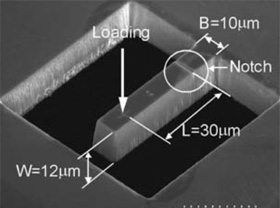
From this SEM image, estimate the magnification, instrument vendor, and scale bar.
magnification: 1.3 K X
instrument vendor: Hitachi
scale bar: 23100 nm